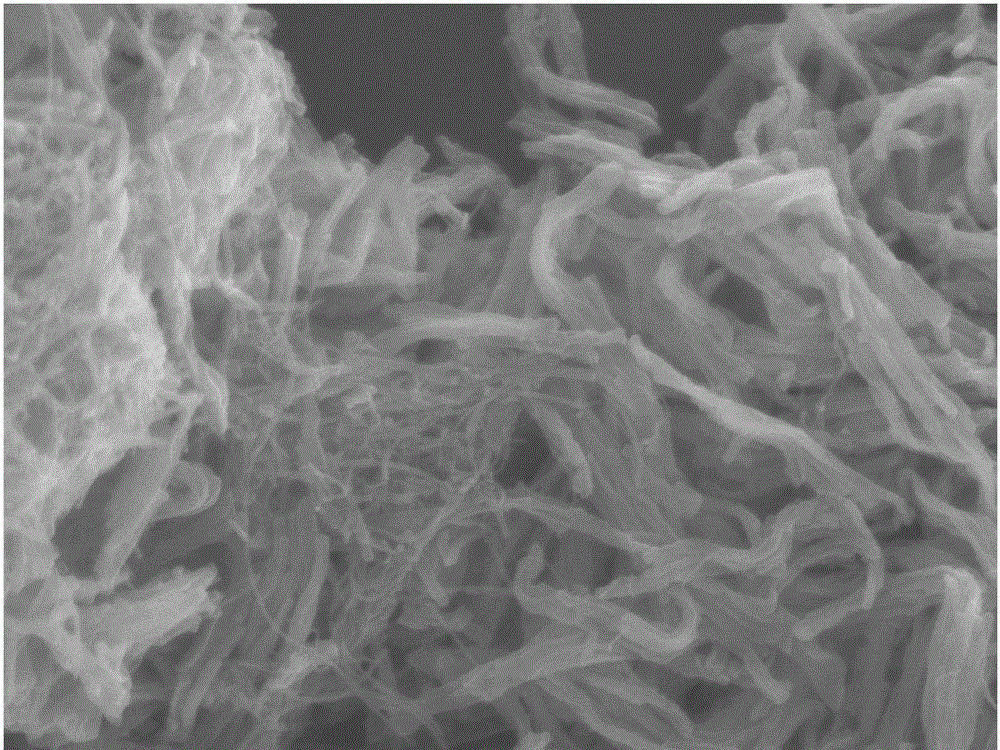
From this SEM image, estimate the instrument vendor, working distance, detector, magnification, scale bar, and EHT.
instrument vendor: JEOL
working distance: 7.9 mm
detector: SEI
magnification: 20 K X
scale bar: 1000 nm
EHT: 8 kV